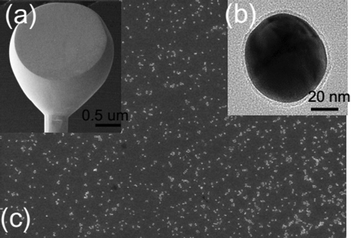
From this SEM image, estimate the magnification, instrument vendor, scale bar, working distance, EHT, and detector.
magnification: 9 K X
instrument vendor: Hitachi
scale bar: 5000 nm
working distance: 7 mm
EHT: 15 kV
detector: SE(U)